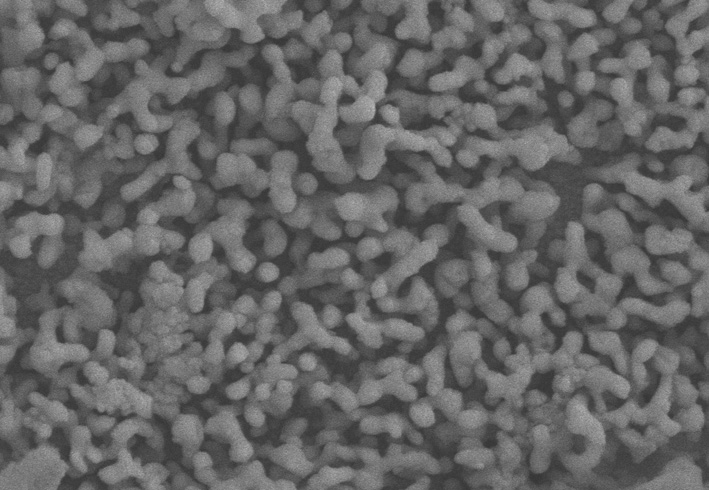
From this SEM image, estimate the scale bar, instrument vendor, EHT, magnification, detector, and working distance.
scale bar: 1000 nm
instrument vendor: Zeiss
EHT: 10 kV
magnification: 10 K X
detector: SE2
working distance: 7.6 mm